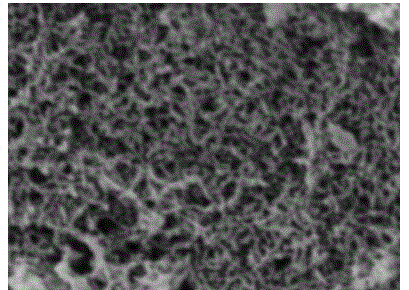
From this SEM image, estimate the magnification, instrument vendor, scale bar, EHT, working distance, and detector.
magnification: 23.4 K X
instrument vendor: Tescan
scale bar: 1000 nm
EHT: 5 kV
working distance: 7.9 mm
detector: SE